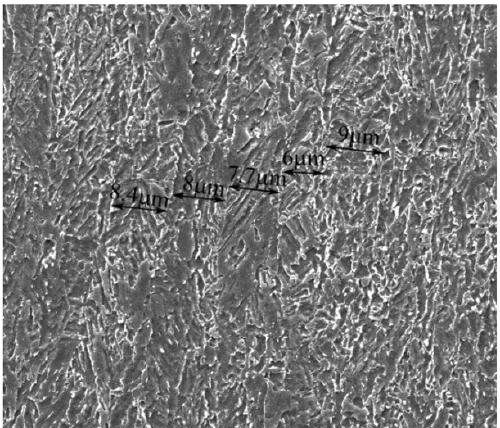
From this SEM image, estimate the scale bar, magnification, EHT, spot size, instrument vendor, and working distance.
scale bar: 20000 nm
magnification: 2 K X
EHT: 20 kV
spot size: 3.5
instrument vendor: FEI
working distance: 10.4 mm